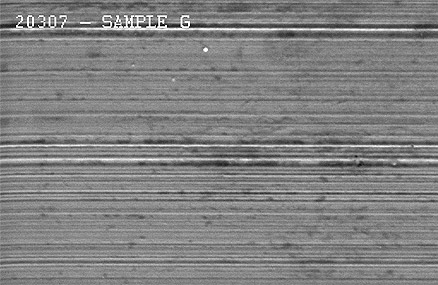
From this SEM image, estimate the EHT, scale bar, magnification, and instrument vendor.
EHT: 20 kV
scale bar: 10000 nm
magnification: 1 K X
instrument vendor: JEOL